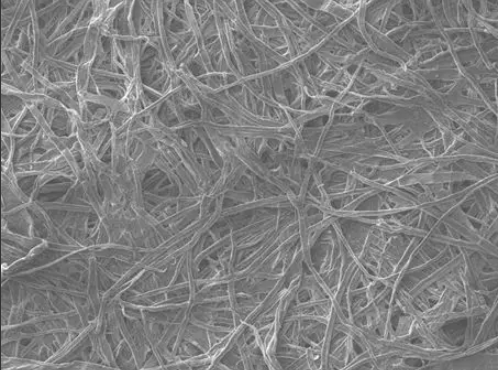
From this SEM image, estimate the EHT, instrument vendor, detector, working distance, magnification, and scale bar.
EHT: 15 kV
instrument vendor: JEOL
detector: LEI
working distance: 7.8 mm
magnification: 0.1 K X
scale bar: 100000 nm